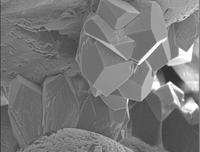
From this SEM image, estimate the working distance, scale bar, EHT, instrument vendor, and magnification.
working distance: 14 mm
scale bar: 10000 nm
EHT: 7 kV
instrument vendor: JEOL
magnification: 1.6 K X